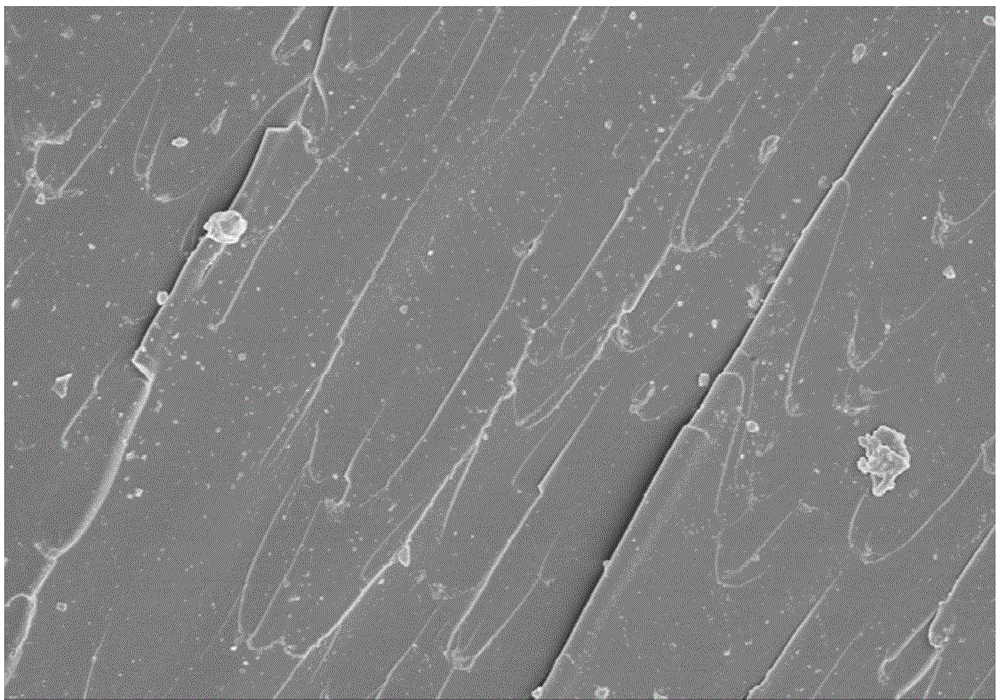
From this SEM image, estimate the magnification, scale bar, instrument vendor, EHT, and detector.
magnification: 1 K X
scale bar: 50000 nm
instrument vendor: Hitachi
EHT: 5 kV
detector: SE(M,LA0)